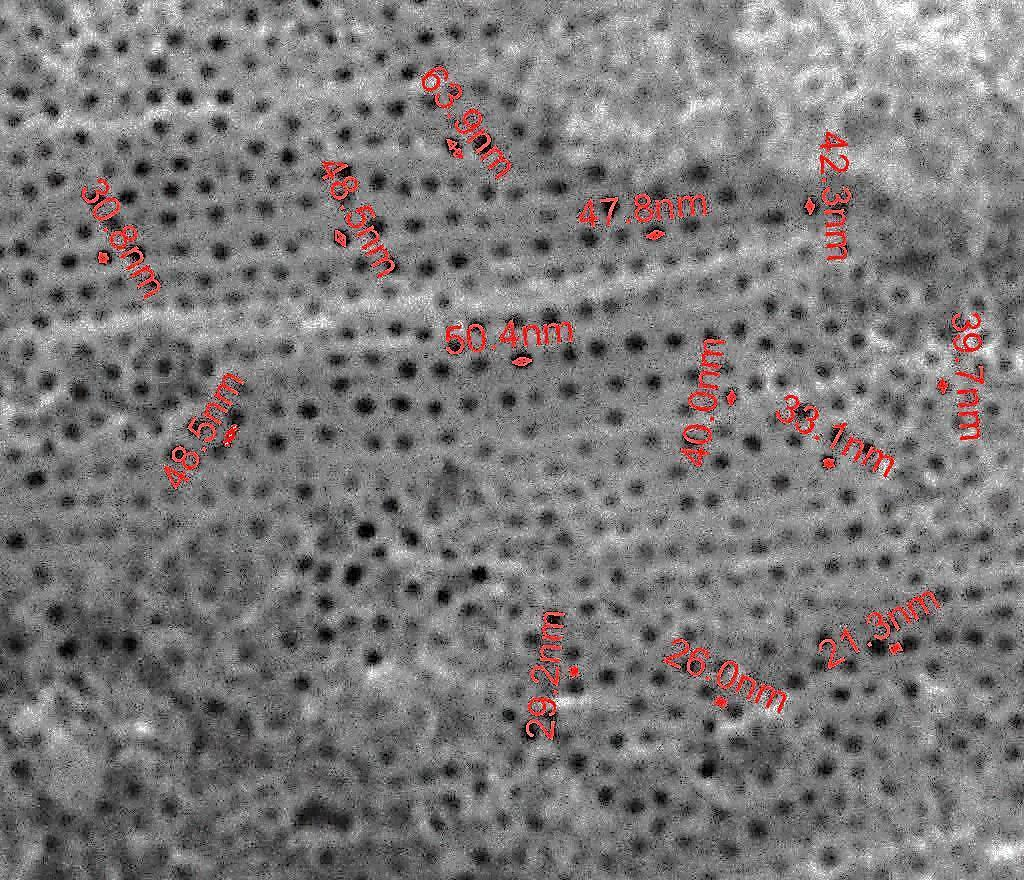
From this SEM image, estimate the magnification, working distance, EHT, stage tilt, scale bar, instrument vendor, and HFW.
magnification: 100 K X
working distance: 10 mm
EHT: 25 kV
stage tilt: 0°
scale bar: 500 nm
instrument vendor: FEI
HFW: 2.7 µm